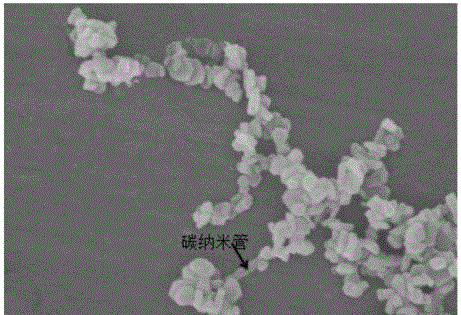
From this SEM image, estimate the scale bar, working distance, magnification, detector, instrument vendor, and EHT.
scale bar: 500 nm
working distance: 9.7 mm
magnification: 70 K X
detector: SE(M)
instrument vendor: Hitachi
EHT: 3 kV